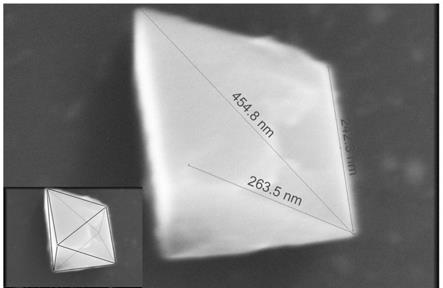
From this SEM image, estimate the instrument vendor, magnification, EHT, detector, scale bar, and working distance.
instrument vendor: FEI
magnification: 650 K X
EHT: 15 kV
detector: TLD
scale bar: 200 nm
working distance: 4.2 mm